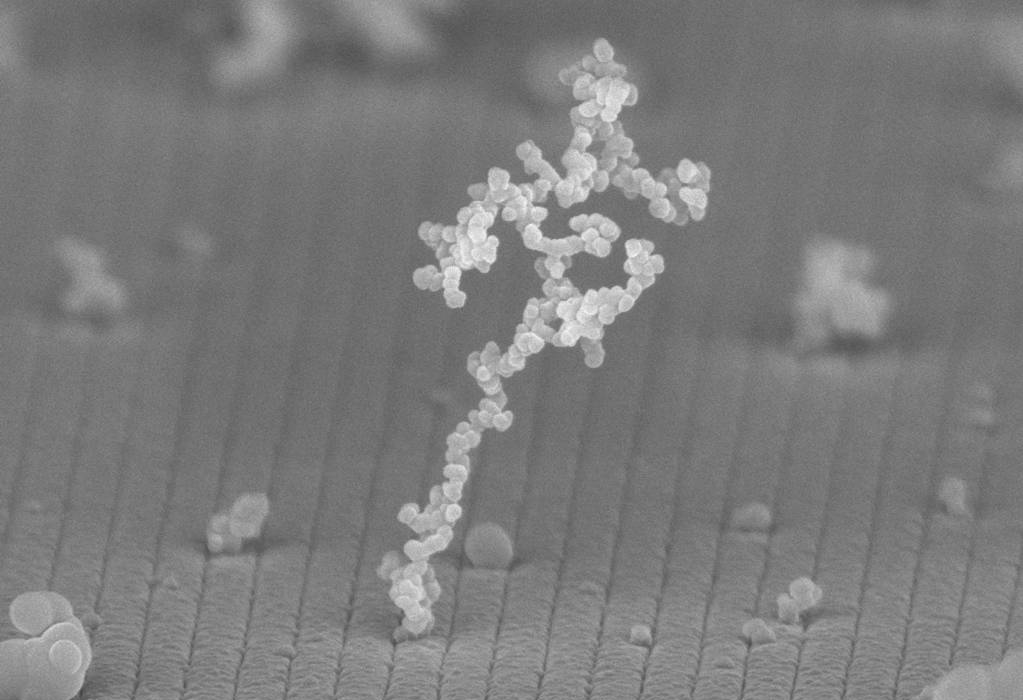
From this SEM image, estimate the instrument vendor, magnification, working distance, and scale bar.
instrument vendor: Zeiss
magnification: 91.06 K X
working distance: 5 mm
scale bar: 300 nm